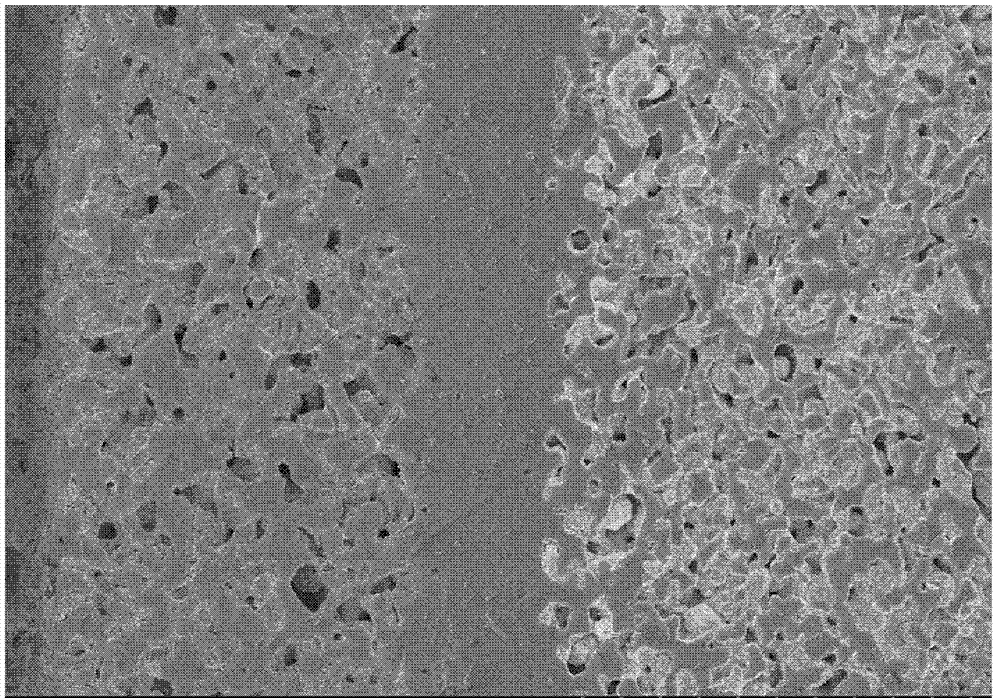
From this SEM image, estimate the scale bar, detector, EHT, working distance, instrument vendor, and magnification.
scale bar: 50000 nm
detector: SE(U)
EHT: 5 kV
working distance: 11 mm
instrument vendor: Hitachi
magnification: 1 K X